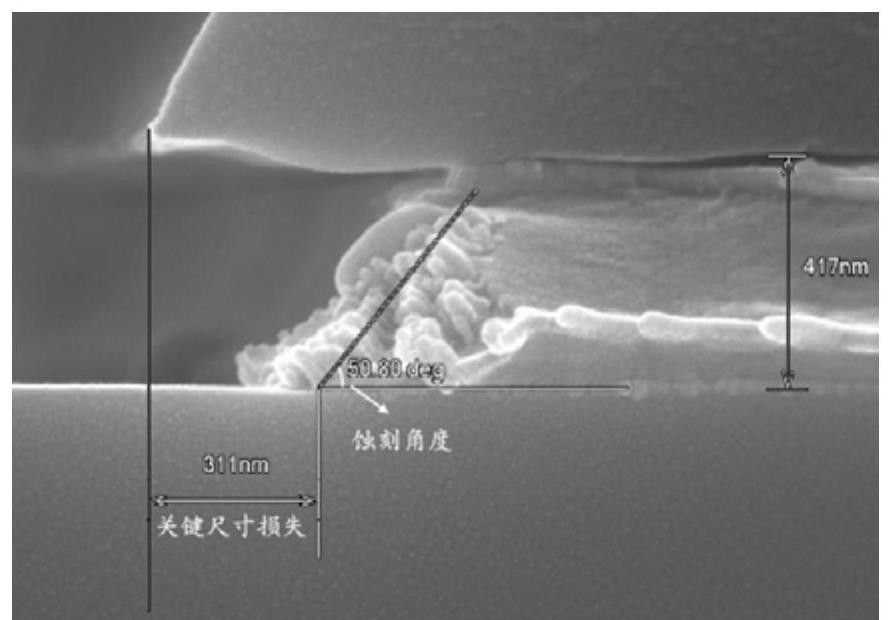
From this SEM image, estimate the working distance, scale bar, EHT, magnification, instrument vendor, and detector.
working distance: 9.1 mm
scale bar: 500 nm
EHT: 10 kV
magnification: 80 K X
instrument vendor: Hitachi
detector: SE(U)